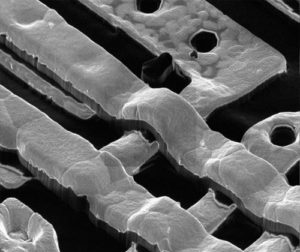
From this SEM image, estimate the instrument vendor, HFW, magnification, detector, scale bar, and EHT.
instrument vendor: FEI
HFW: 15.2 µm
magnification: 20 K X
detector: CDM-E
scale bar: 2000 nm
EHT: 30 kV